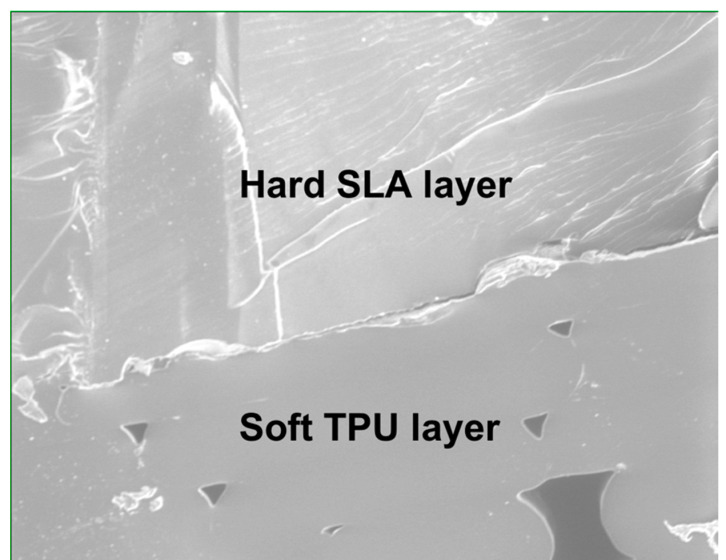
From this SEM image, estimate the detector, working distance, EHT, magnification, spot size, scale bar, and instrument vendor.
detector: SE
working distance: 11 mm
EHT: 10 kV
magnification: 0.324 K X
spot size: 3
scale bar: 400000 nm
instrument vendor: FEI